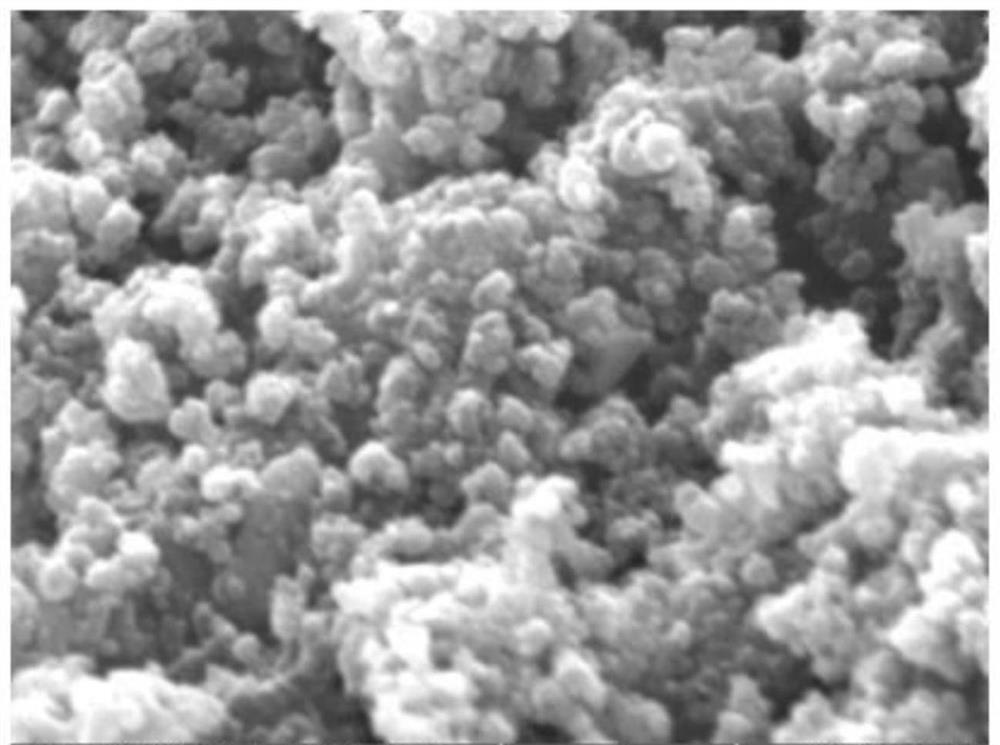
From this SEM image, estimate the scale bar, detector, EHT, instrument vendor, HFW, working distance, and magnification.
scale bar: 1000 nm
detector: SE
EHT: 30 kV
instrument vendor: Tescan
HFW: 4.15 µm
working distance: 6.97 mm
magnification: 50 K X